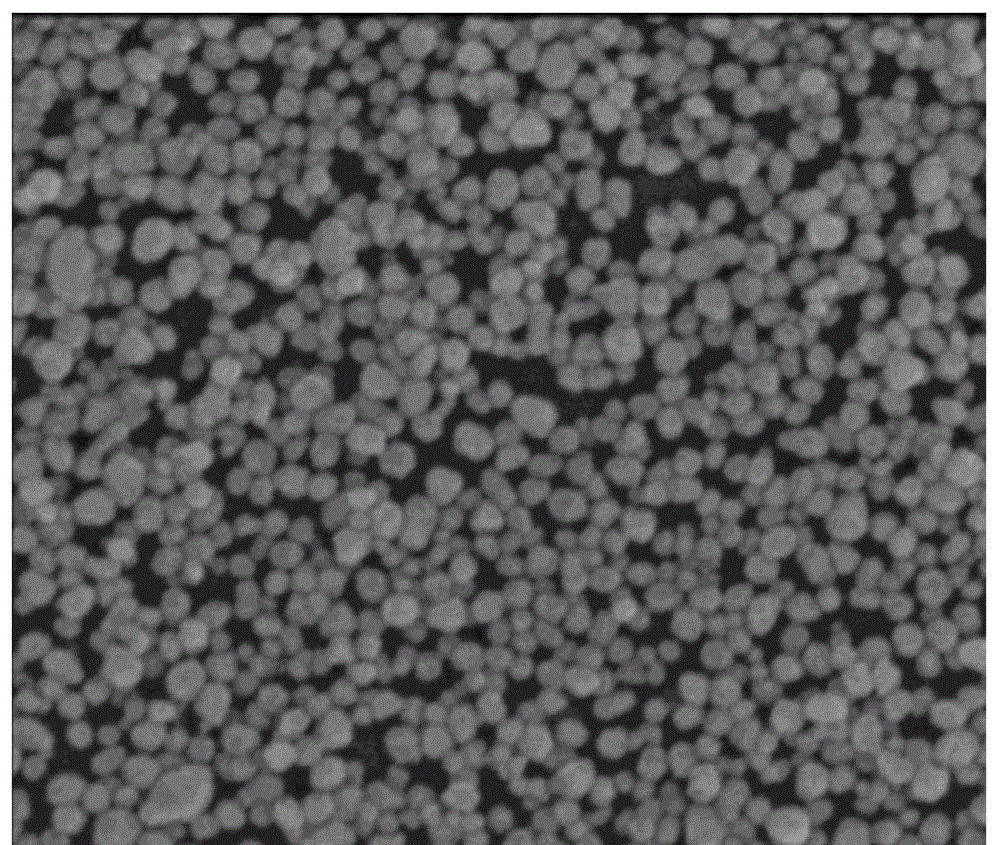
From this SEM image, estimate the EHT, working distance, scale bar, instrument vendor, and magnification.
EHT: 5 kV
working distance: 4.8 mm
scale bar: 500 nm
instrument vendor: FEI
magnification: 100 K X